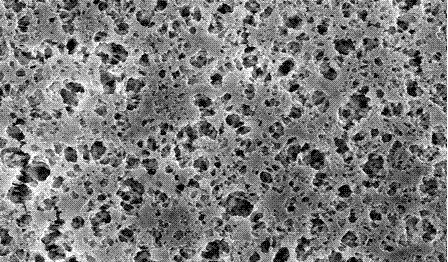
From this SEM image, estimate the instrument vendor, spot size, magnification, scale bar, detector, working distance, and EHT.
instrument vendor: FEI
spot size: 4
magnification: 2 K X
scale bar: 20000 nm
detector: SE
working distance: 7.4 mm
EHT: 25 kV